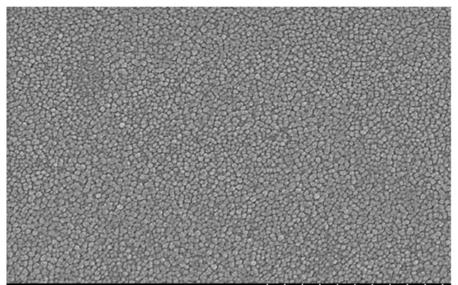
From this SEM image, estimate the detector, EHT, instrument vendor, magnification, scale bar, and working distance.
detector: SE(M)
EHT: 15 kV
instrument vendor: Hitachi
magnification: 100 K X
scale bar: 500 nm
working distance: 9.3 mm